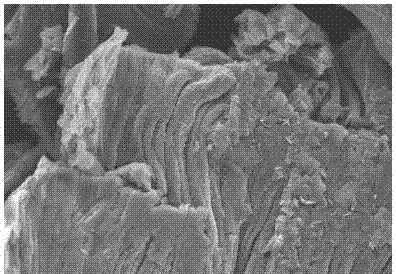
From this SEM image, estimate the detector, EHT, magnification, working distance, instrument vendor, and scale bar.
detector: SE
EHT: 15 kV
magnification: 1.1 K X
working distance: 10.1 mm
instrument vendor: Hitachi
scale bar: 50000 nm